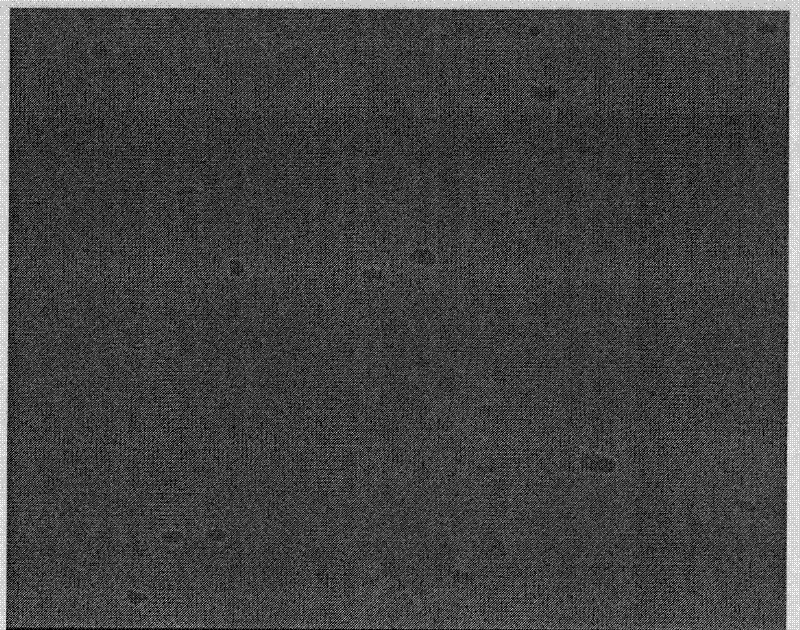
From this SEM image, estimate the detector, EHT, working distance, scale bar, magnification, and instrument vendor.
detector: SE(M)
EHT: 10 kV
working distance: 13.4 mm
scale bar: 1000 nm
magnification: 30 K X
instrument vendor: Hitachi